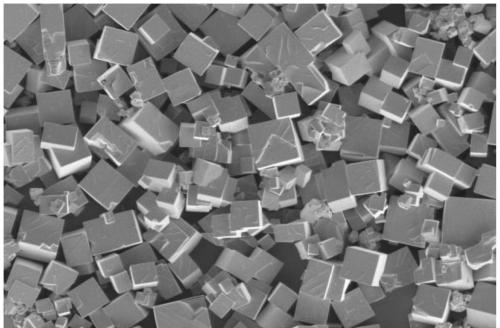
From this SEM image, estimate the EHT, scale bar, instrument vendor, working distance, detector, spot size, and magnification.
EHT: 3 kV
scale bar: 40000 nm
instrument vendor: FEI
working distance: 2.9 mm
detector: ETD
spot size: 4.5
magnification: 5 K X